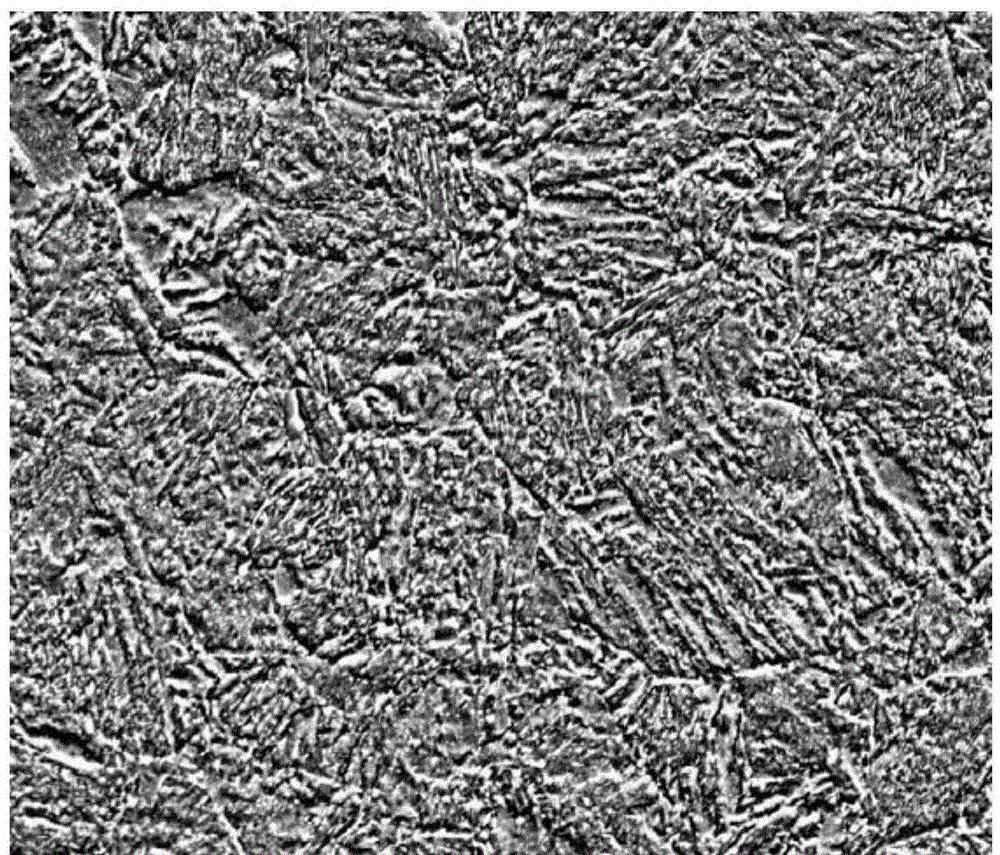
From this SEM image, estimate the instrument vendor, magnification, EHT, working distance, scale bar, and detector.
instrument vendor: FEI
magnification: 5 K X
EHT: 15 kV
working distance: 5 mm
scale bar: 20000 nm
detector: ETD